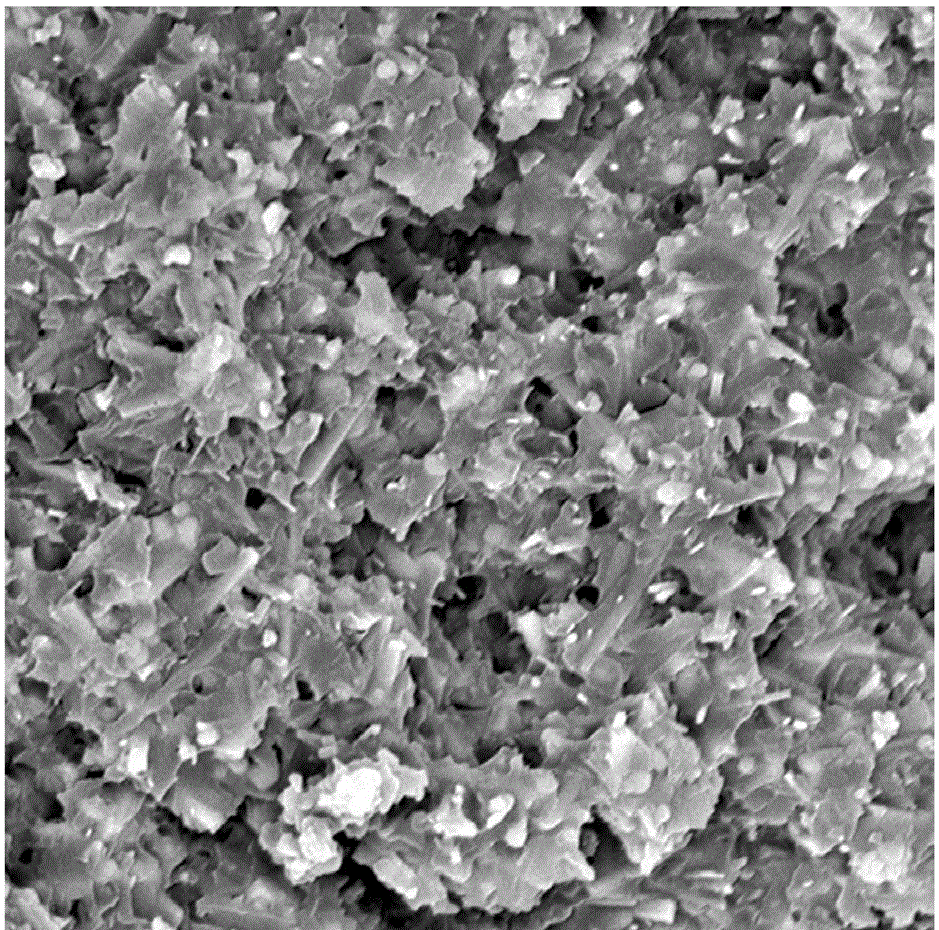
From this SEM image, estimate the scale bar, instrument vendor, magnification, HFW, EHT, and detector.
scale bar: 5000 nm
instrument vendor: other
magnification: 11.5 K X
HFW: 23.3 µm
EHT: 15 kV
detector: BSD Full